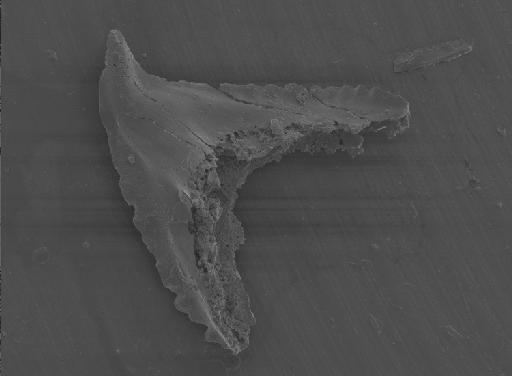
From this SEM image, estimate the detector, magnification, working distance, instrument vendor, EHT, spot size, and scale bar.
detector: SE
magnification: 0.15 K X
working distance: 10.1 mm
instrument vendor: FEI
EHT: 5 kV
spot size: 3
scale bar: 500000 nm